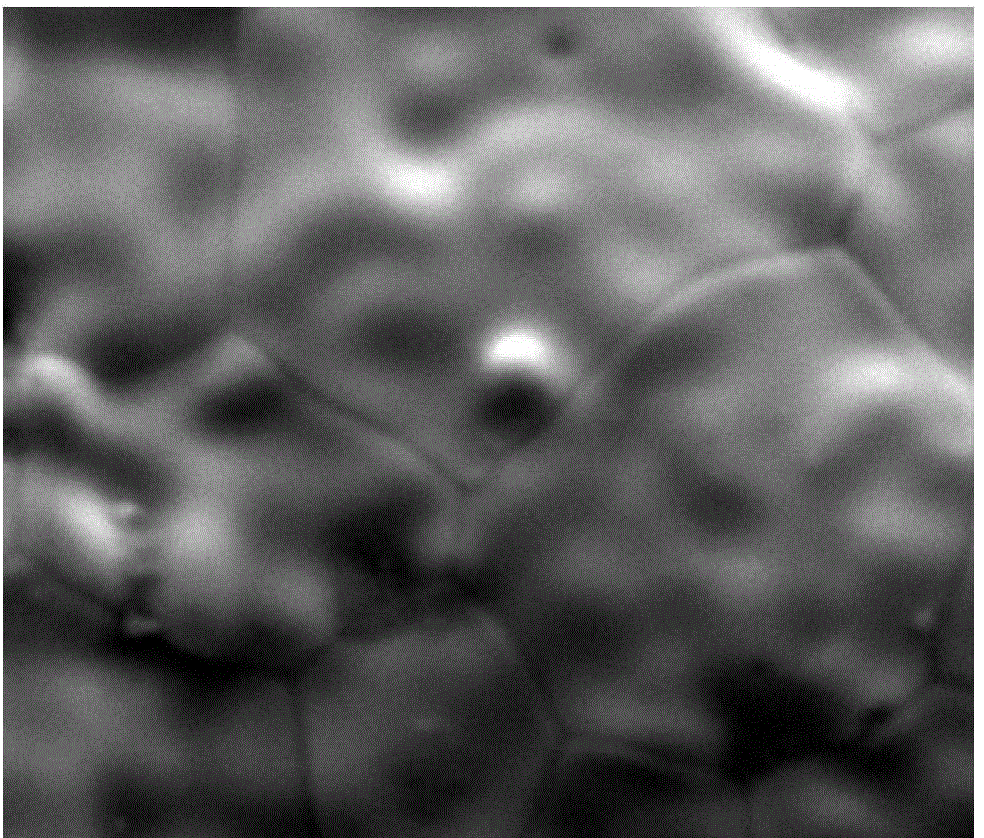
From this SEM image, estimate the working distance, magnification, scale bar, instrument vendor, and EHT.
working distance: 13.1 mm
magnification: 2.5 K X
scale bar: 20000 nm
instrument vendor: FEI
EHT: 20 kV